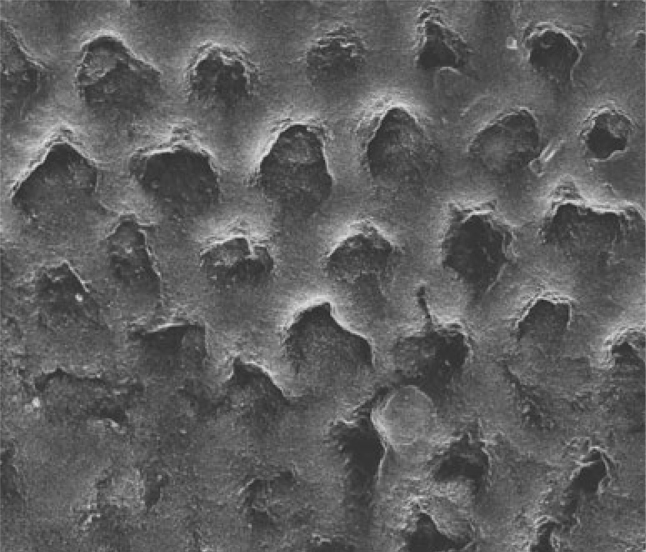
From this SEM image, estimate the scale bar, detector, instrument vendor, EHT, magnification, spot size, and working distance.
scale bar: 20000 nm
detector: ETD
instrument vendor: FEI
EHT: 20 kV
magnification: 6 K X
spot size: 2.5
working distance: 10.9 mm